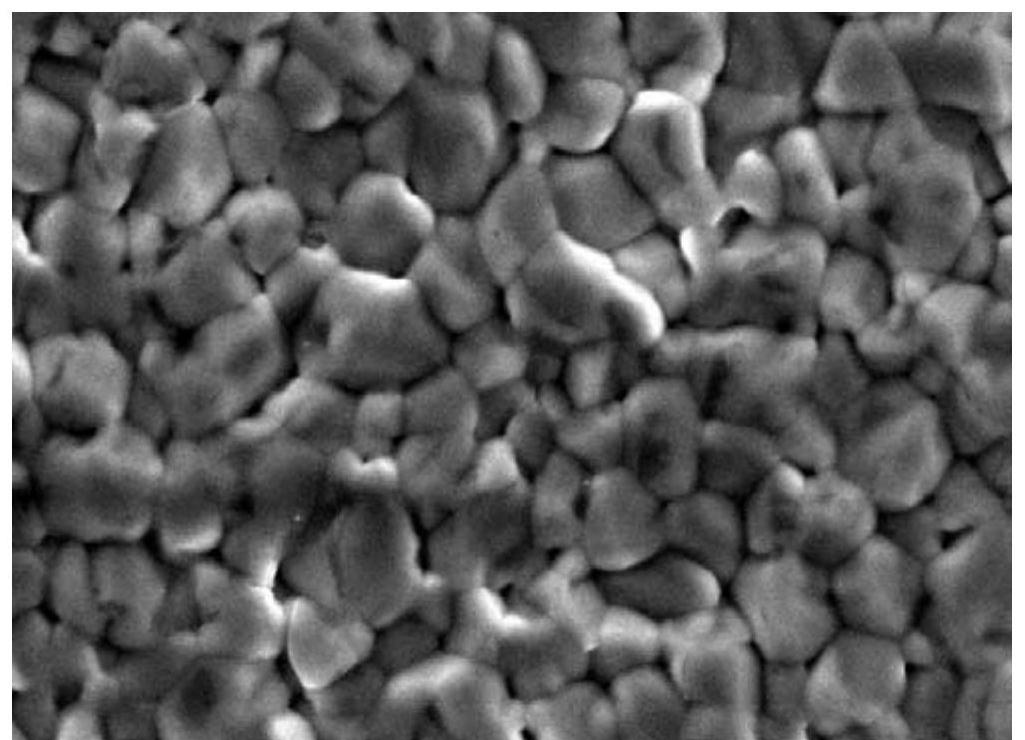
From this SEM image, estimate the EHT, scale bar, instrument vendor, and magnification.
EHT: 20 kV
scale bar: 10000 nm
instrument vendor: JEOL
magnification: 3.5 K X